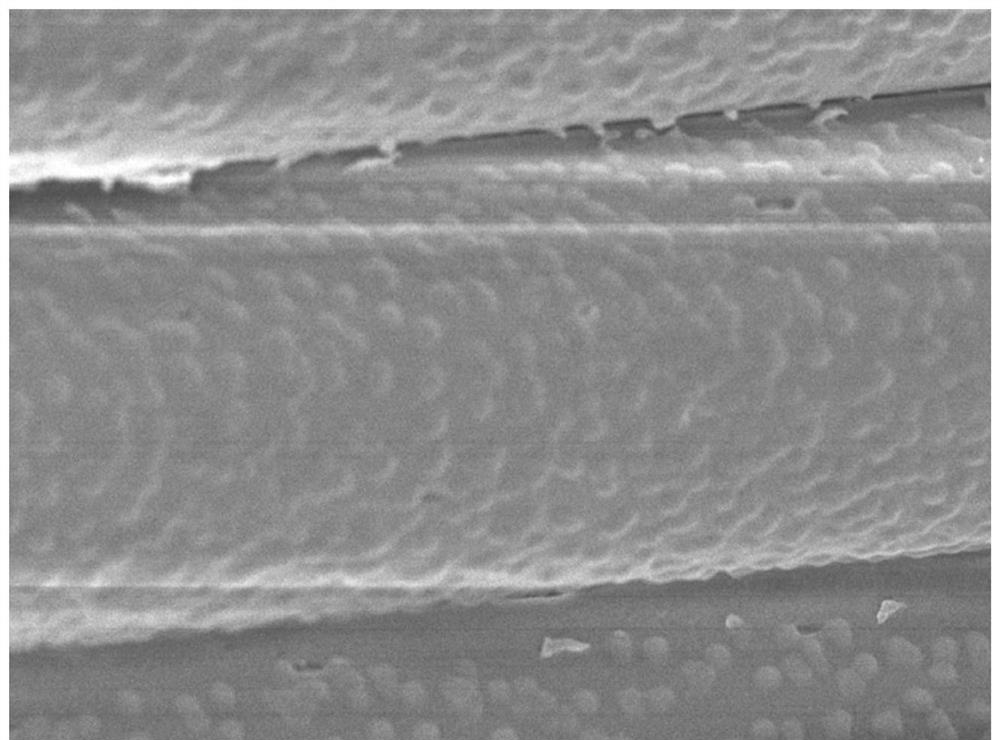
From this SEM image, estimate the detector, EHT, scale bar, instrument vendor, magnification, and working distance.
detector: SEI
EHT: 5 kV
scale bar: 1000 nm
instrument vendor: JEOL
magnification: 5.5 K X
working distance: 8 mm